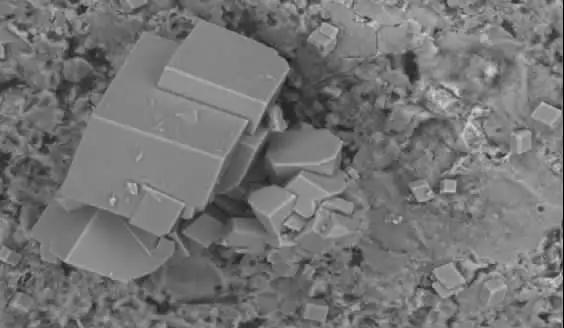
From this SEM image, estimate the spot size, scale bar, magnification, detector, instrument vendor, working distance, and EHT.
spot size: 4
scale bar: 50000 nm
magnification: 0.5 K X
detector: BSE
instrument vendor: FEI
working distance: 10.2 mm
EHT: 20 kV